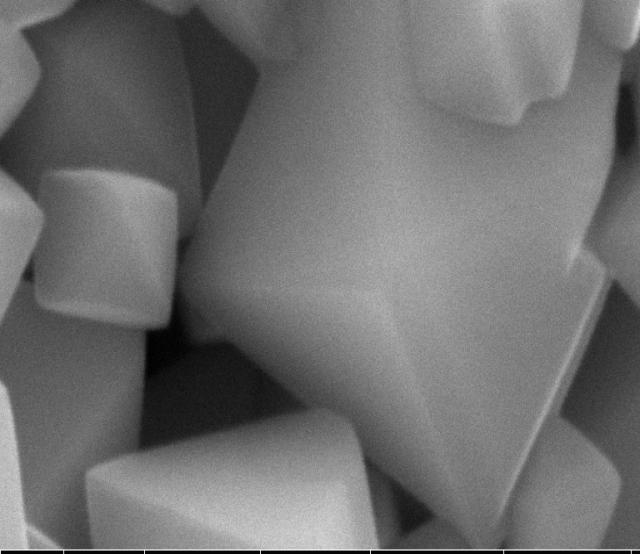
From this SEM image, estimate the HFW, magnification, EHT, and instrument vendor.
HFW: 4.14 µm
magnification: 100 K X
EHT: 15 kV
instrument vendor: FEI